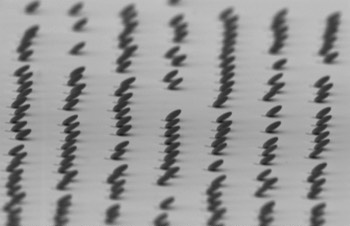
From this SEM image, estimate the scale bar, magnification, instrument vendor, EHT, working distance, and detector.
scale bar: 1000 nm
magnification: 6.89 K X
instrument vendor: Zeiss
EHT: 3 kV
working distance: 11 mm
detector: SE2